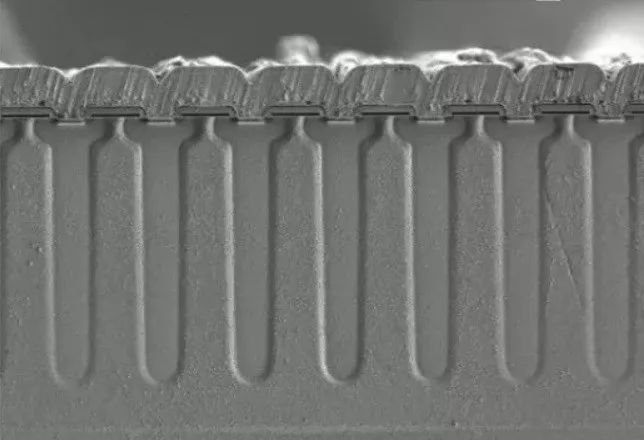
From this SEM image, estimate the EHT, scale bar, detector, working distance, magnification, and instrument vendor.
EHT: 1 kV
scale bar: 40000 nm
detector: SE(L)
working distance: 10.2 mm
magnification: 1.3 K X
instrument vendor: Hitachi